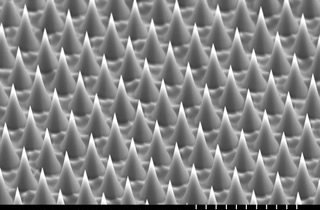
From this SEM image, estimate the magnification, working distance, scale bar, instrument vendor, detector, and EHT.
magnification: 2 K X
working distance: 12.4 mm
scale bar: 20000 nm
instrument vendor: Hitachi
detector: SE(L)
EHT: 15 kV